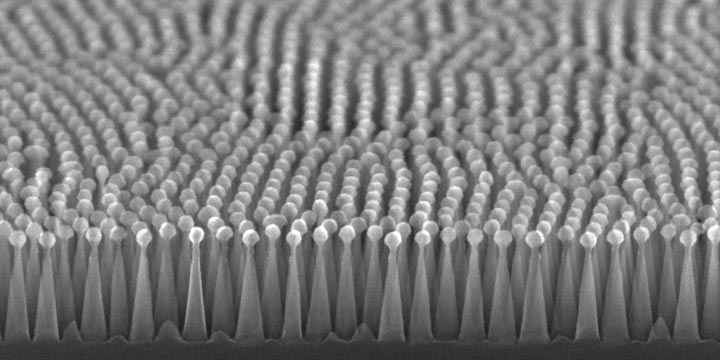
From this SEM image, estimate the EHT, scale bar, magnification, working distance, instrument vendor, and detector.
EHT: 20 kV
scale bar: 500 nm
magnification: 100 K X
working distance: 5.7 mm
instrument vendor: Hitachi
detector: SE(U,LA0)